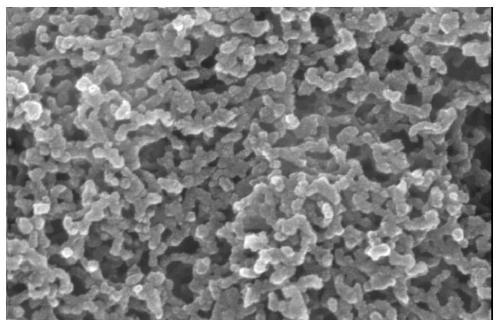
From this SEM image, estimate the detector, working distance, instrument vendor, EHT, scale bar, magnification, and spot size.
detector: TLD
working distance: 6.7 mm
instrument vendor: FEI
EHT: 20 kV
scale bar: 200 nm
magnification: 200 K X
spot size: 4